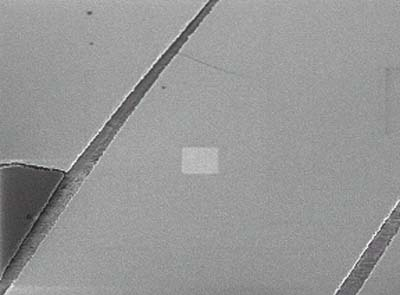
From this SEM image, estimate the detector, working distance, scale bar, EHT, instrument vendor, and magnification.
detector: SEI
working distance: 3 mm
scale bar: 1000 nm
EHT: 1 kV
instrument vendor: JEOL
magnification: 10 K X